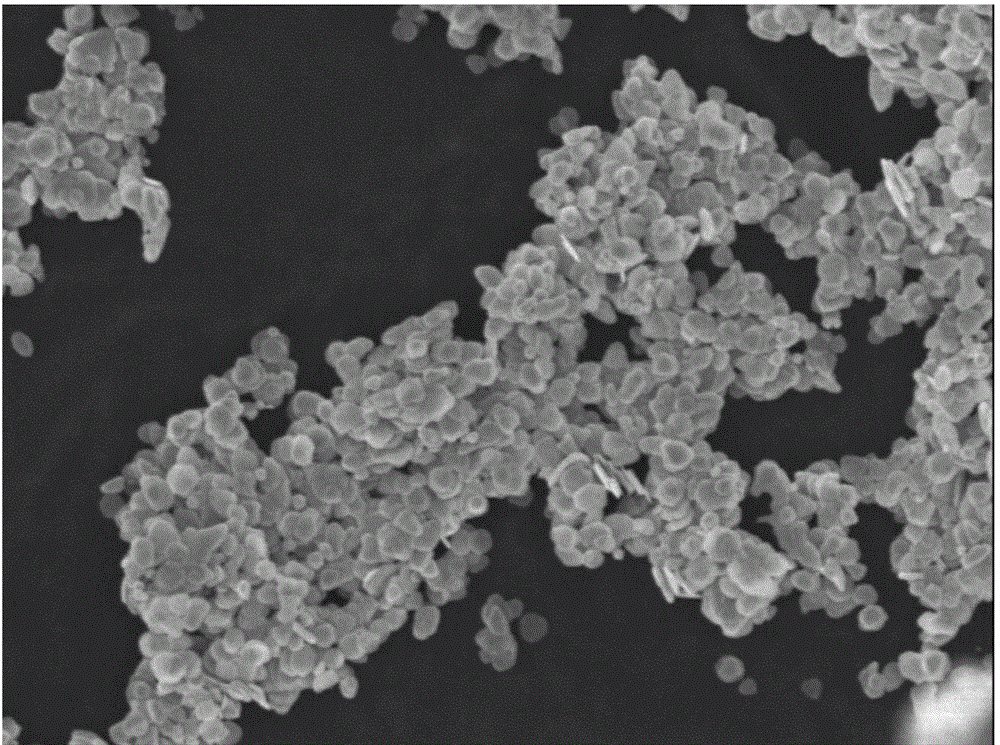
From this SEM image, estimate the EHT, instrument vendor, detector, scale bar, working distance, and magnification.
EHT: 5 kV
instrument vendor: JEOL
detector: SEI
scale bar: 1000 nm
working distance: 8.2 mm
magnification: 10 K X